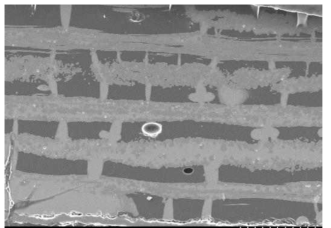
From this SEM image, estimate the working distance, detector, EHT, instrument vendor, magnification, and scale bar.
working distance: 11.6 mm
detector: SE(U)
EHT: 20 kV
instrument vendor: Hitachi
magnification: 0.06 K X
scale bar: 500000 nm